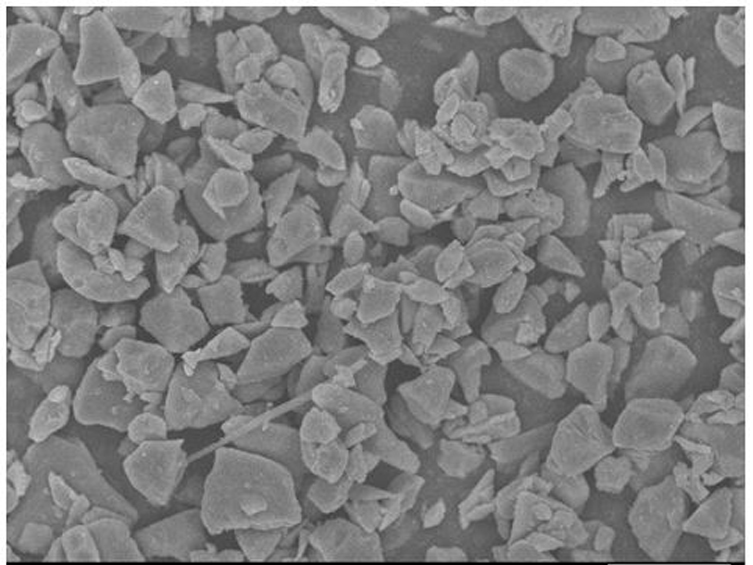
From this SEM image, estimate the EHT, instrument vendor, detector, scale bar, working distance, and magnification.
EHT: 10 kV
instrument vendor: JEOL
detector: SEI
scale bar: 10000 nm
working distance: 7.8 mm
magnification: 2 K X